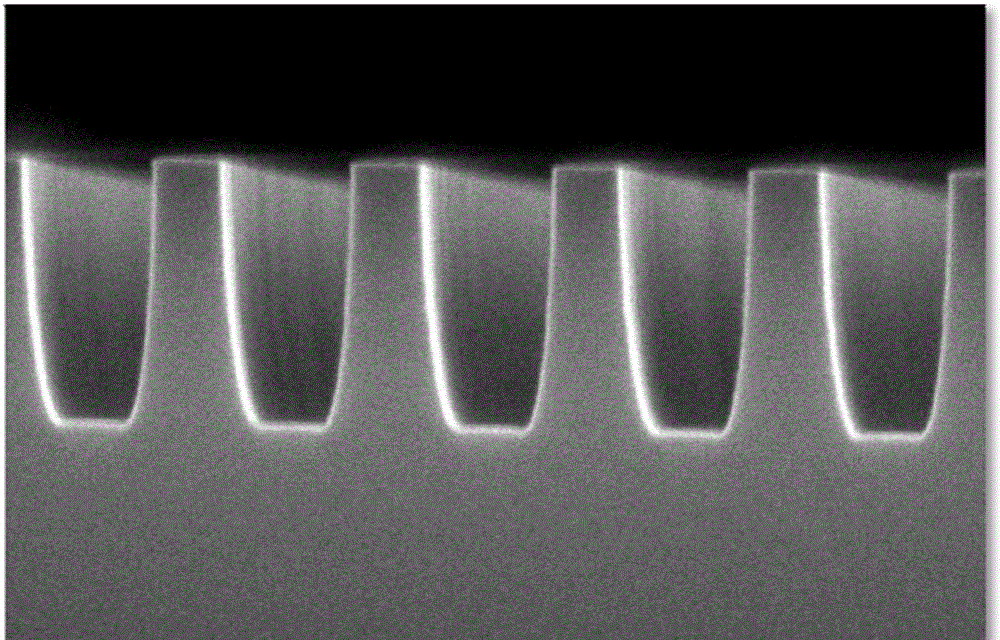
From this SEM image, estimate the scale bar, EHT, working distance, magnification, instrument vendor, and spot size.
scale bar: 500 nm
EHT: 10 kV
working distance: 4.9 mm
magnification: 152.563 K X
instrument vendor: FEI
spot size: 4